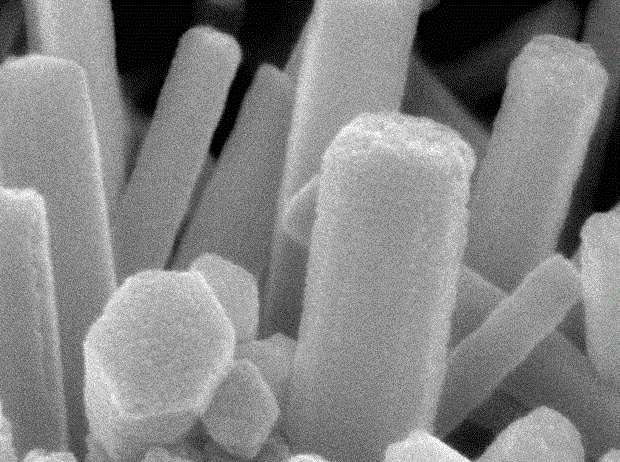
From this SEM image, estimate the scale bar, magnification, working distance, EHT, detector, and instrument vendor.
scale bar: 100 nm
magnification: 150 K X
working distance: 7.1 mm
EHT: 8 kV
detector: SEI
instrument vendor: JEOL